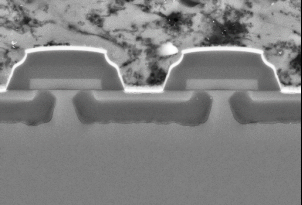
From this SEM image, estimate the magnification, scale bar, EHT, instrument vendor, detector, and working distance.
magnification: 10 K X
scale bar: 2000 nm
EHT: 10 kV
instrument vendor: Zeiss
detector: BSD1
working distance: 7.3 mm